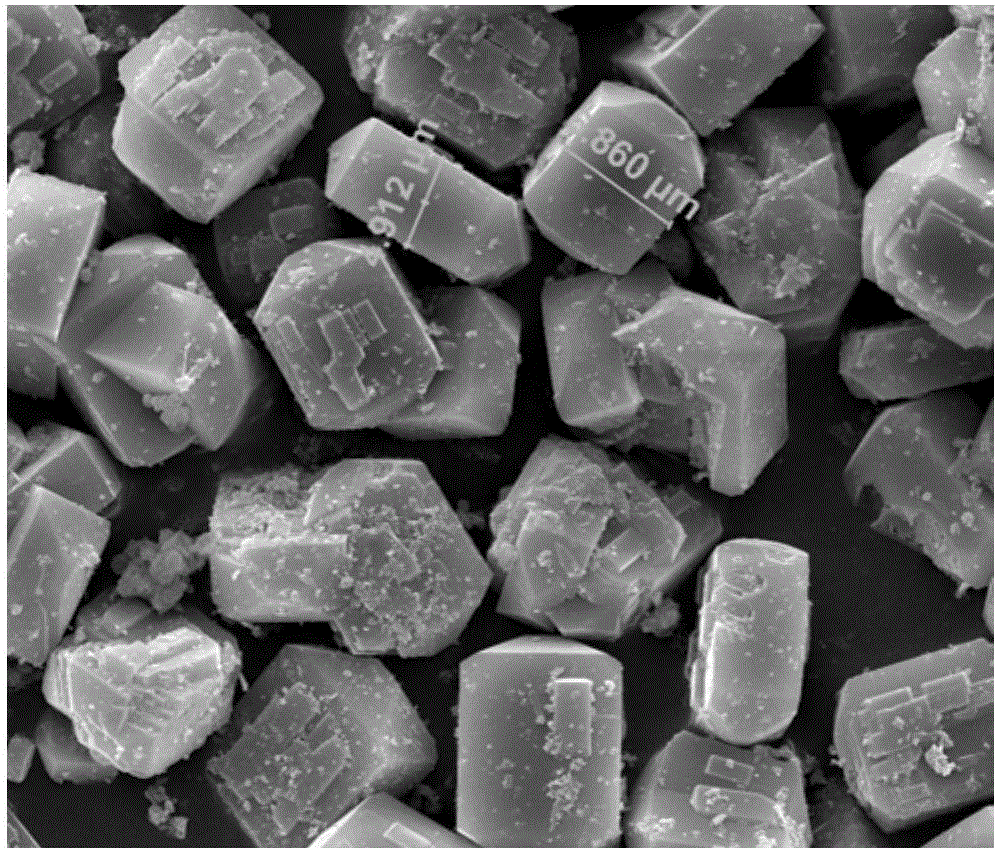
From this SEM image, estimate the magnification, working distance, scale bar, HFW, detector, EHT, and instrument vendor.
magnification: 5 K X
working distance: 15.2 mm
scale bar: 20000 nm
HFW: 51.2 µm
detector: ETD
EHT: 20 kV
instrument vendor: FEI